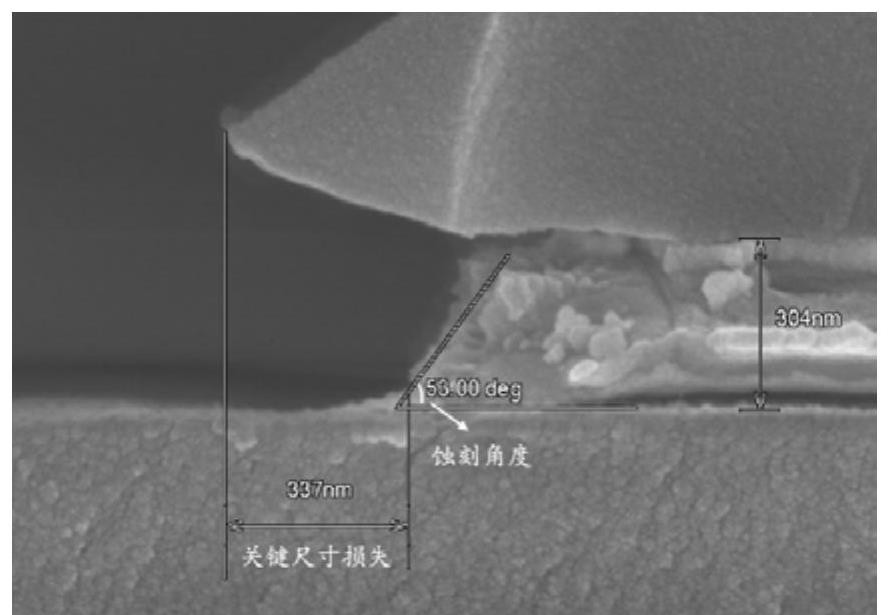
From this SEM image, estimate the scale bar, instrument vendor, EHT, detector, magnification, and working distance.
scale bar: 500 nm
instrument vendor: Hitachi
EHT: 5 kV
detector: SE(U)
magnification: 80 K X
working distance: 9 mm